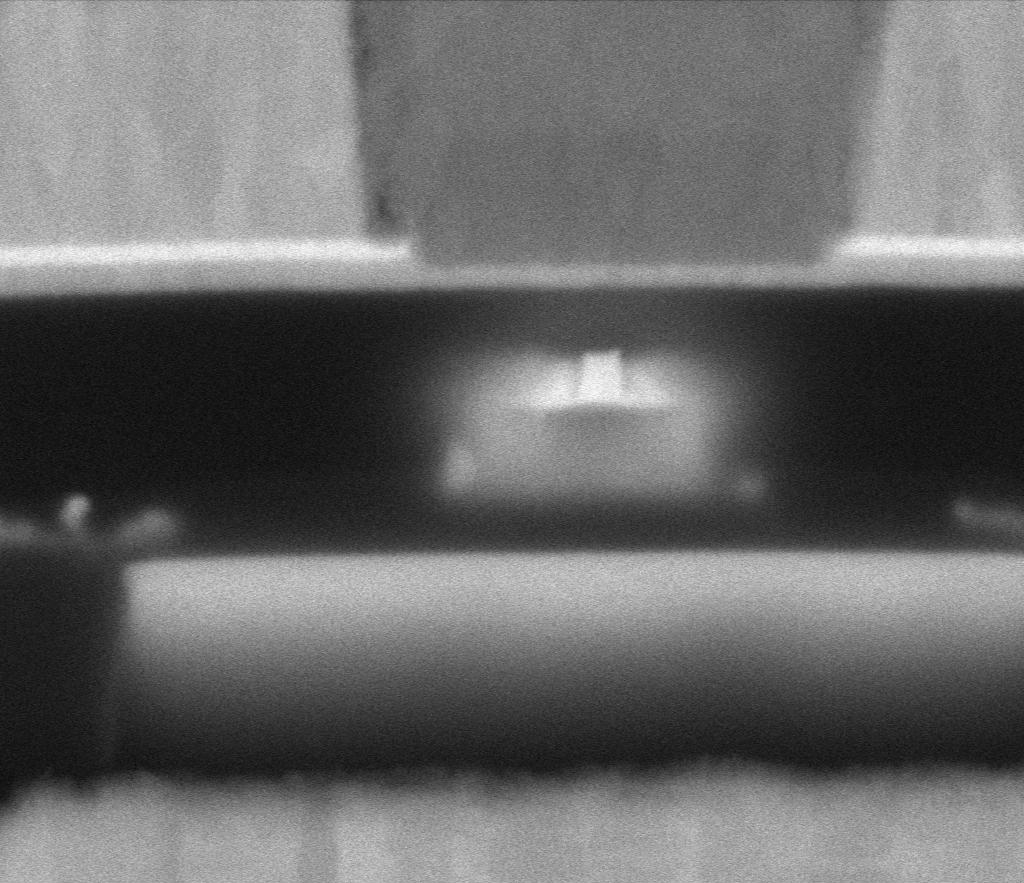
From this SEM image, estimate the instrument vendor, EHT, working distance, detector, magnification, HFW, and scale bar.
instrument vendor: Thermo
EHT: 5 kV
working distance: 2 mm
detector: MD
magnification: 568.609 K X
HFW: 0.635 µm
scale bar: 100 nm